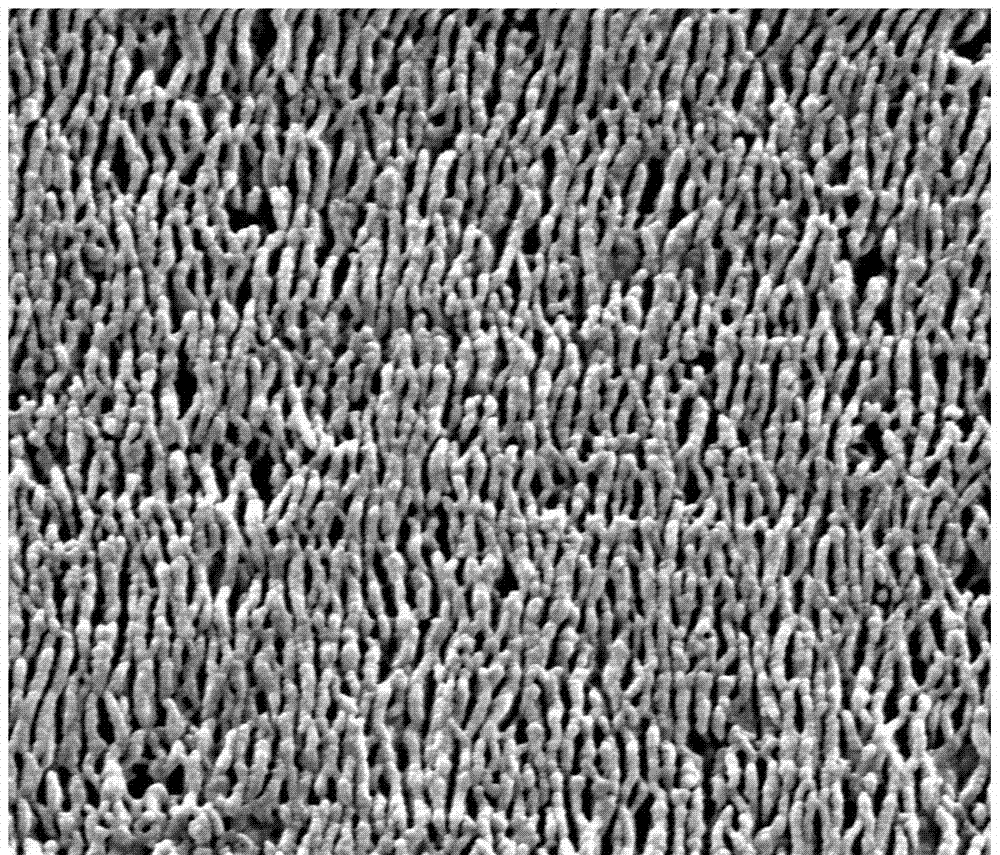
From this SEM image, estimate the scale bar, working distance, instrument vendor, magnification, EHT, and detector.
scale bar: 1000 nm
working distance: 10.6 mm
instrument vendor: FEI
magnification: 80 K X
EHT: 20 kV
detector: SE